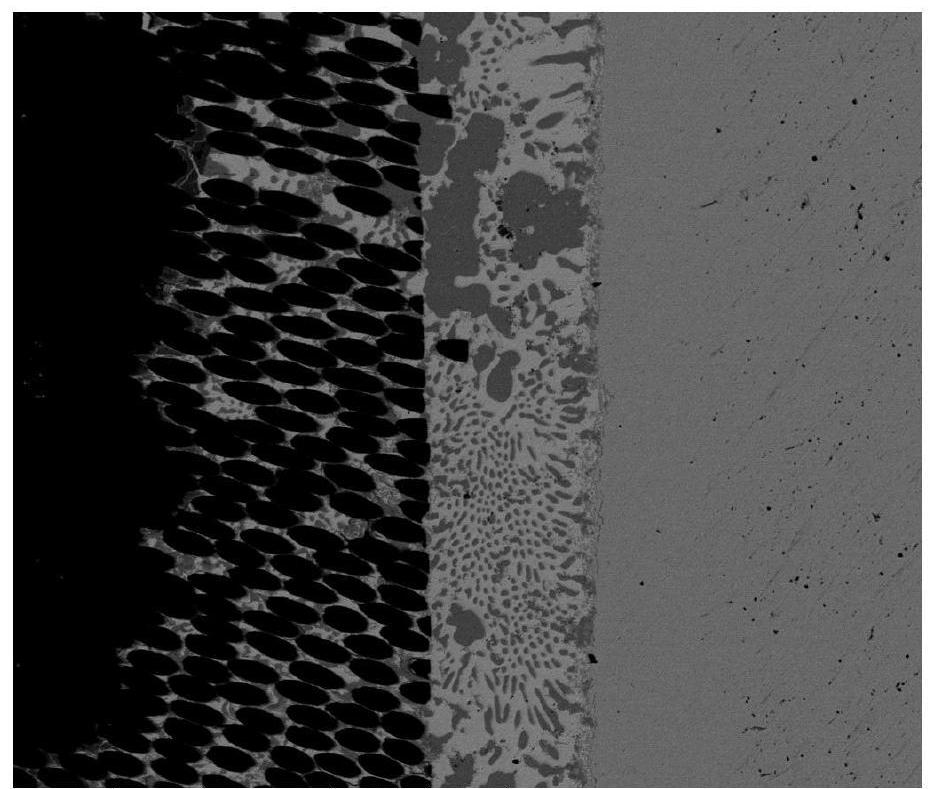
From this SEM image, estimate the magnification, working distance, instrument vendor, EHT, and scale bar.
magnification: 1 K X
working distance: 11.5 mm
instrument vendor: FEI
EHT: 20 kV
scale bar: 100000 nm